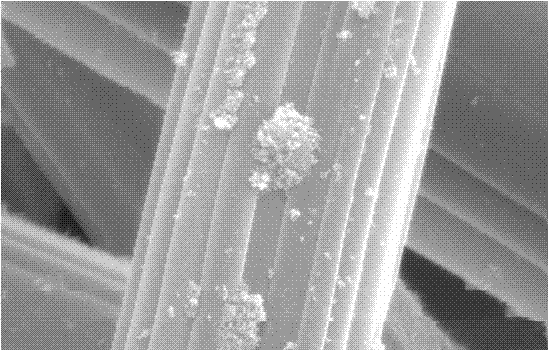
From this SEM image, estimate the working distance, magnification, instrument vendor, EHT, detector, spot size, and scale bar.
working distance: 6.9 mm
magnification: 10 K X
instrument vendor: FEI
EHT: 25 kV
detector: SE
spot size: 4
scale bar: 5000 nm